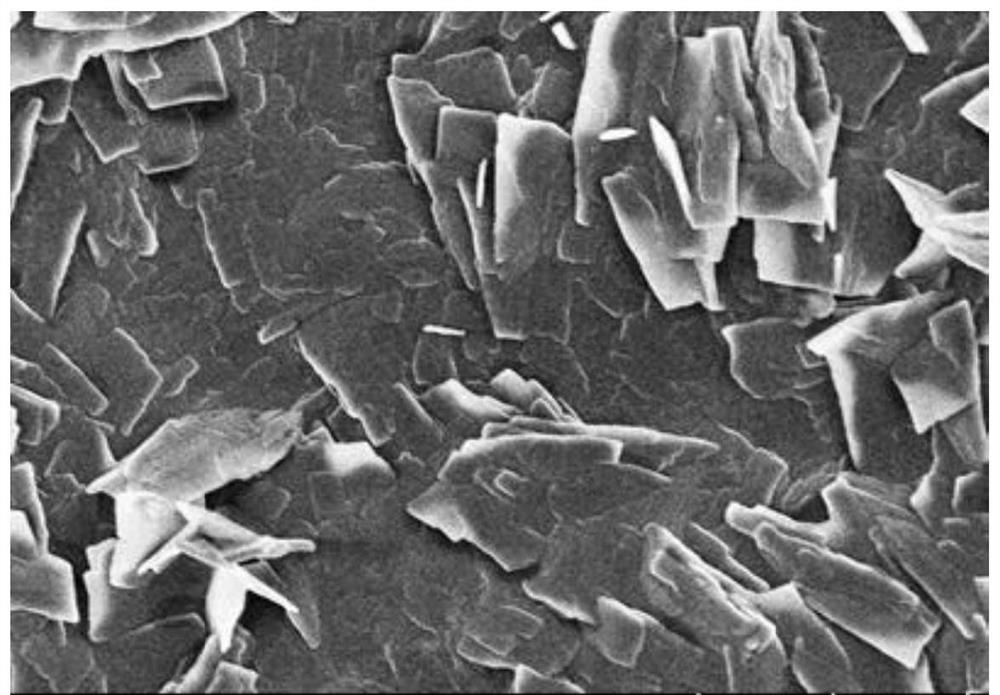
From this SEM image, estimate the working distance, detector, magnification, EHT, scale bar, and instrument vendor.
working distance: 6.7 mm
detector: SE(UL)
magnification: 7 K X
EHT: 3 kV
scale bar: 5000 nm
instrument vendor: Hitachi